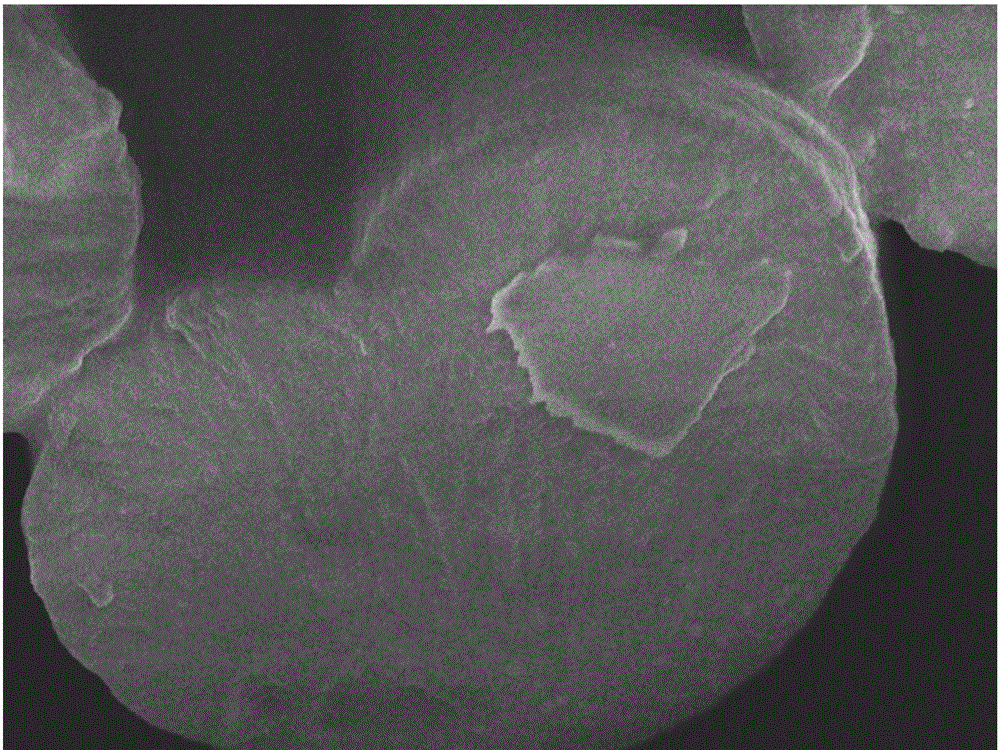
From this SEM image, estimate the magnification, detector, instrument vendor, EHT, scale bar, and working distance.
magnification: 10 K X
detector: SEI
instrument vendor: JEOL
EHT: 3 kV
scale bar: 1000 nm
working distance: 5.8 mm